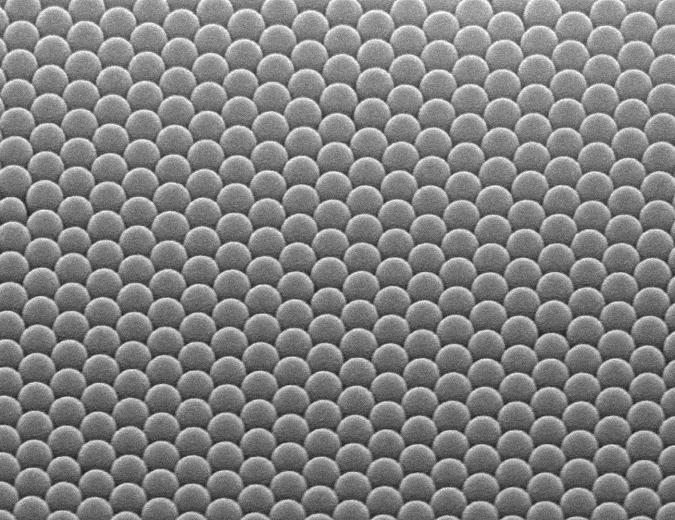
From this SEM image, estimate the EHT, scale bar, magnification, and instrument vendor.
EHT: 15 kV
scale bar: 1000 nm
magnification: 30 K X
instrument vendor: Hitachi